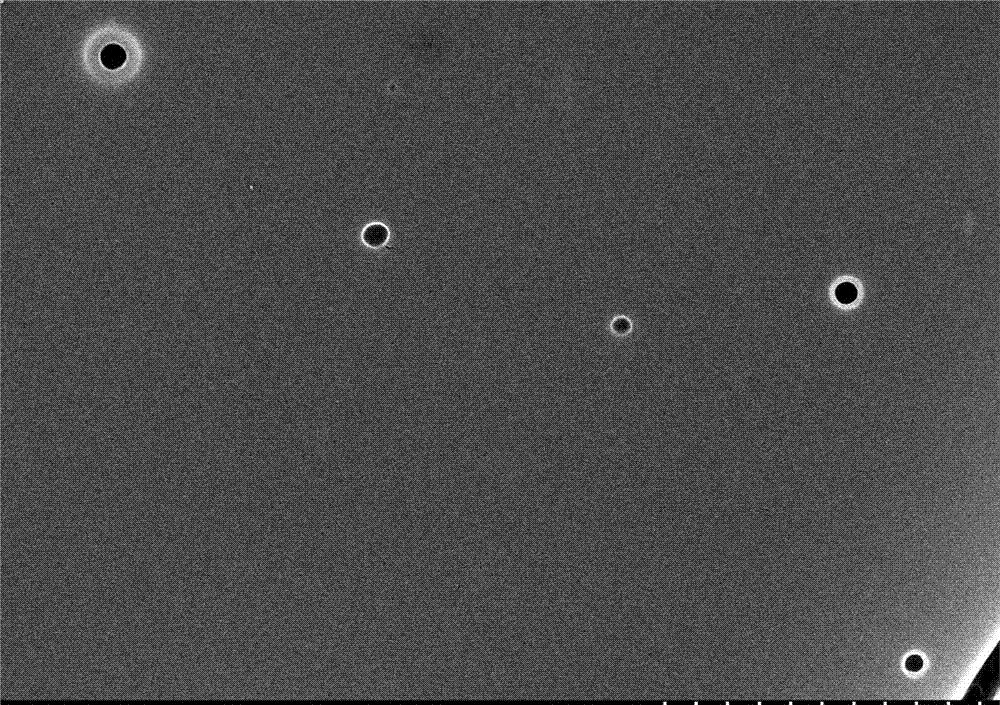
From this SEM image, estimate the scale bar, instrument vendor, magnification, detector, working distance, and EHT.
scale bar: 2000 nm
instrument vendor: Hitachi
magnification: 20 K X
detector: SE(M)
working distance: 8.3 mm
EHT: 10 kV